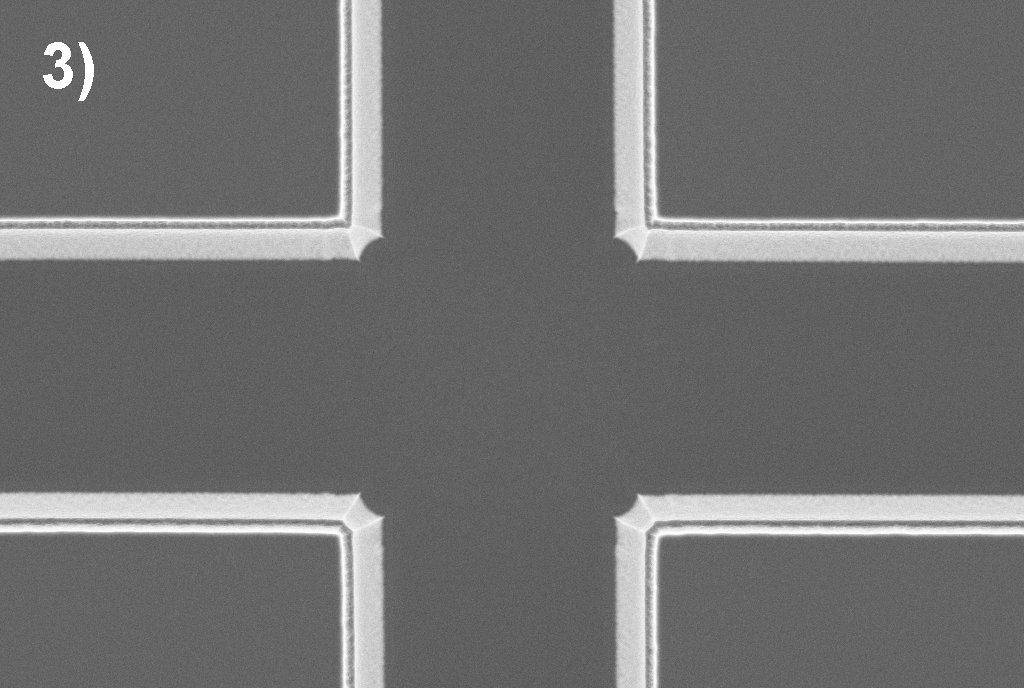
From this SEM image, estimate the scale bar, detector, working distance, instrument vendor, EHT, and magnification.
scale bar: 2000 nm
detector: InLens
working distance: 4.5 mm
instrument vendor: Zeiss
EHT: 5 kV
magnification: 2.2 K X